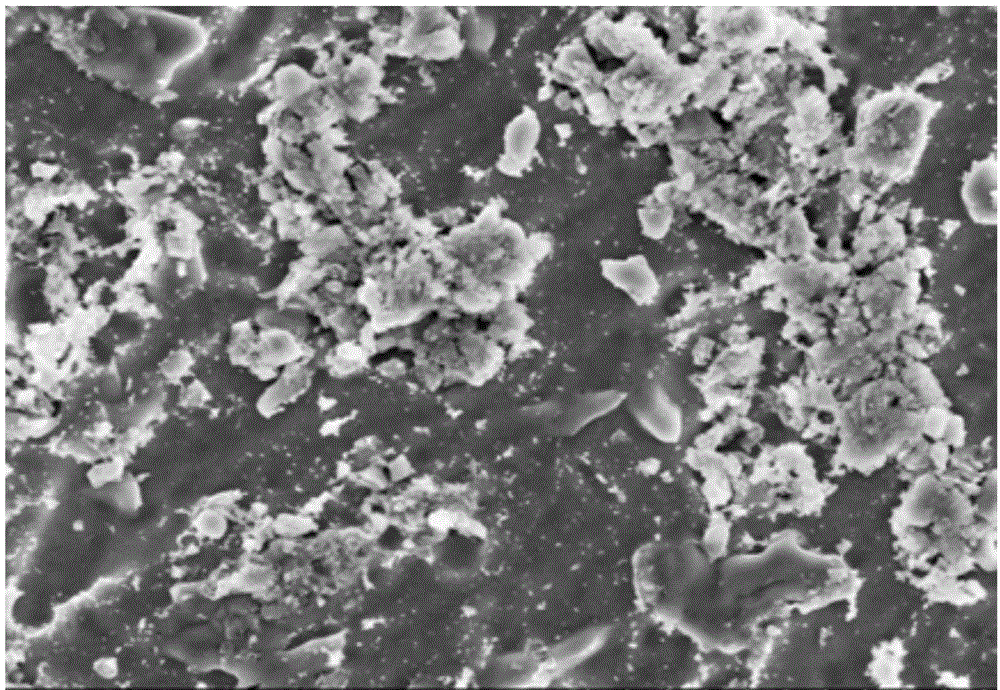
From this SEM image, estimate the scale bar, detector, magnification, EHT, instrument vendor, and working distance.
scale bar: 5000 nm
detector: SE(M,LA0)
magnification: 10 K X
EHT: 5 kV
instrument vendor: Hitachi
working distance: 9.8 mm